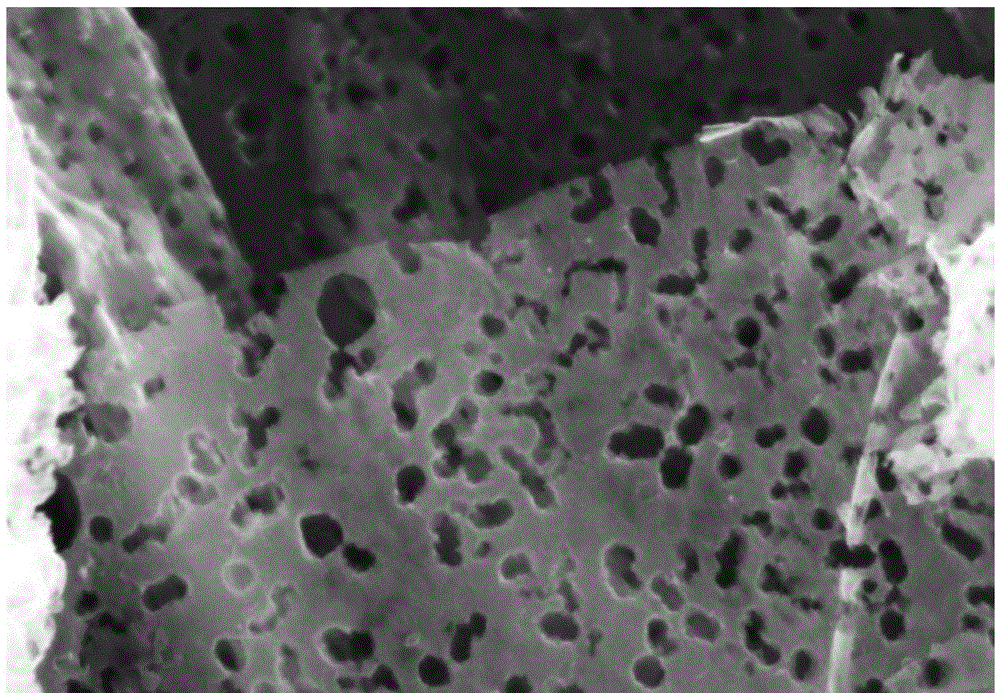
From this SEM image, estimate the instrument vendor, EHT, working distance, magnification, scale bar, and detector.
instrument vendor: Zeiss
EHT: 5 kV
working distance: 8.3 mm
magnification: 50 K X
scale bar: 100 nm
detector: InLens